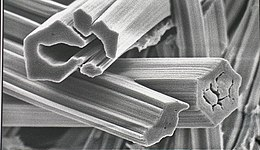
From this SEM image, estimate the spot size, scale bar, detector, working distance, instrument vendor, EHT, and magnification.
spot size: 2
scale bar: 2000 nm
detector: SE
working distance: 4.7 mm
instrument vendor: FEI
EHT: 5 kV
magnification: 12 K X